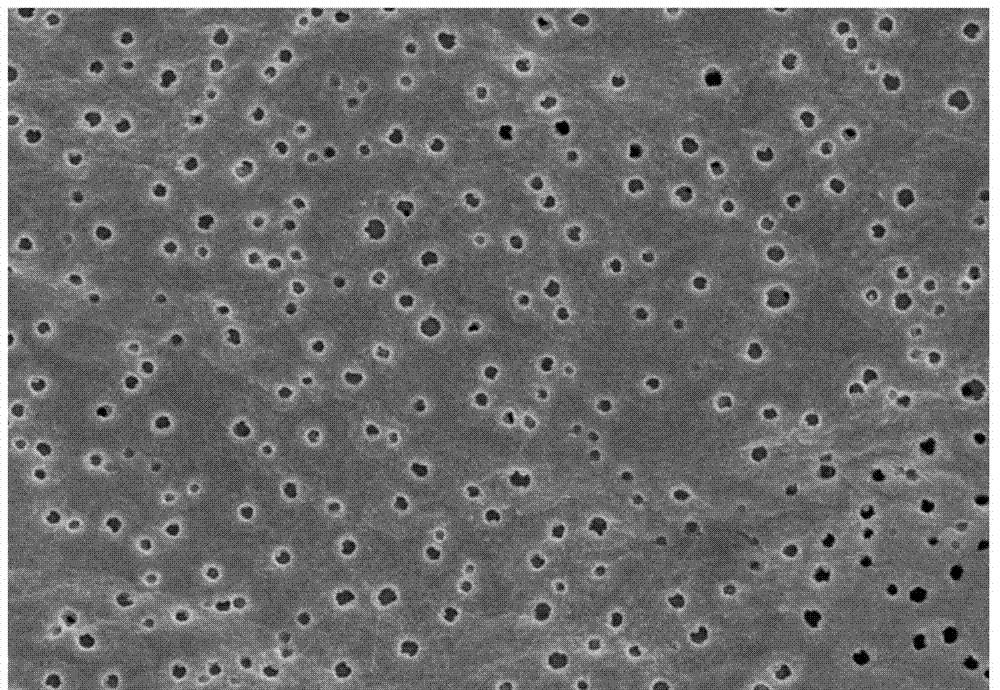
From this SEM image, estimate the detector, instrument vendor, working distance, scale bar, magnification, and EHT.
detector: SE(M)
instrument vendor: Hitachi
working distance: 8 mm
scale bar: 10000 nm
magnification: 5 K X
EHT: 5 kV